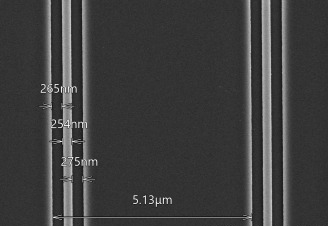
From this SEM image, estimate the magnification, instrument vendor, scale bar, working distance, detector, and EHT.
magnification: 15 K X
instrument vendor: Hitachi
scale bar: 3000 nm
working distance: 12.1 mm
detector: UD(+LD)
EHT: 3 kV